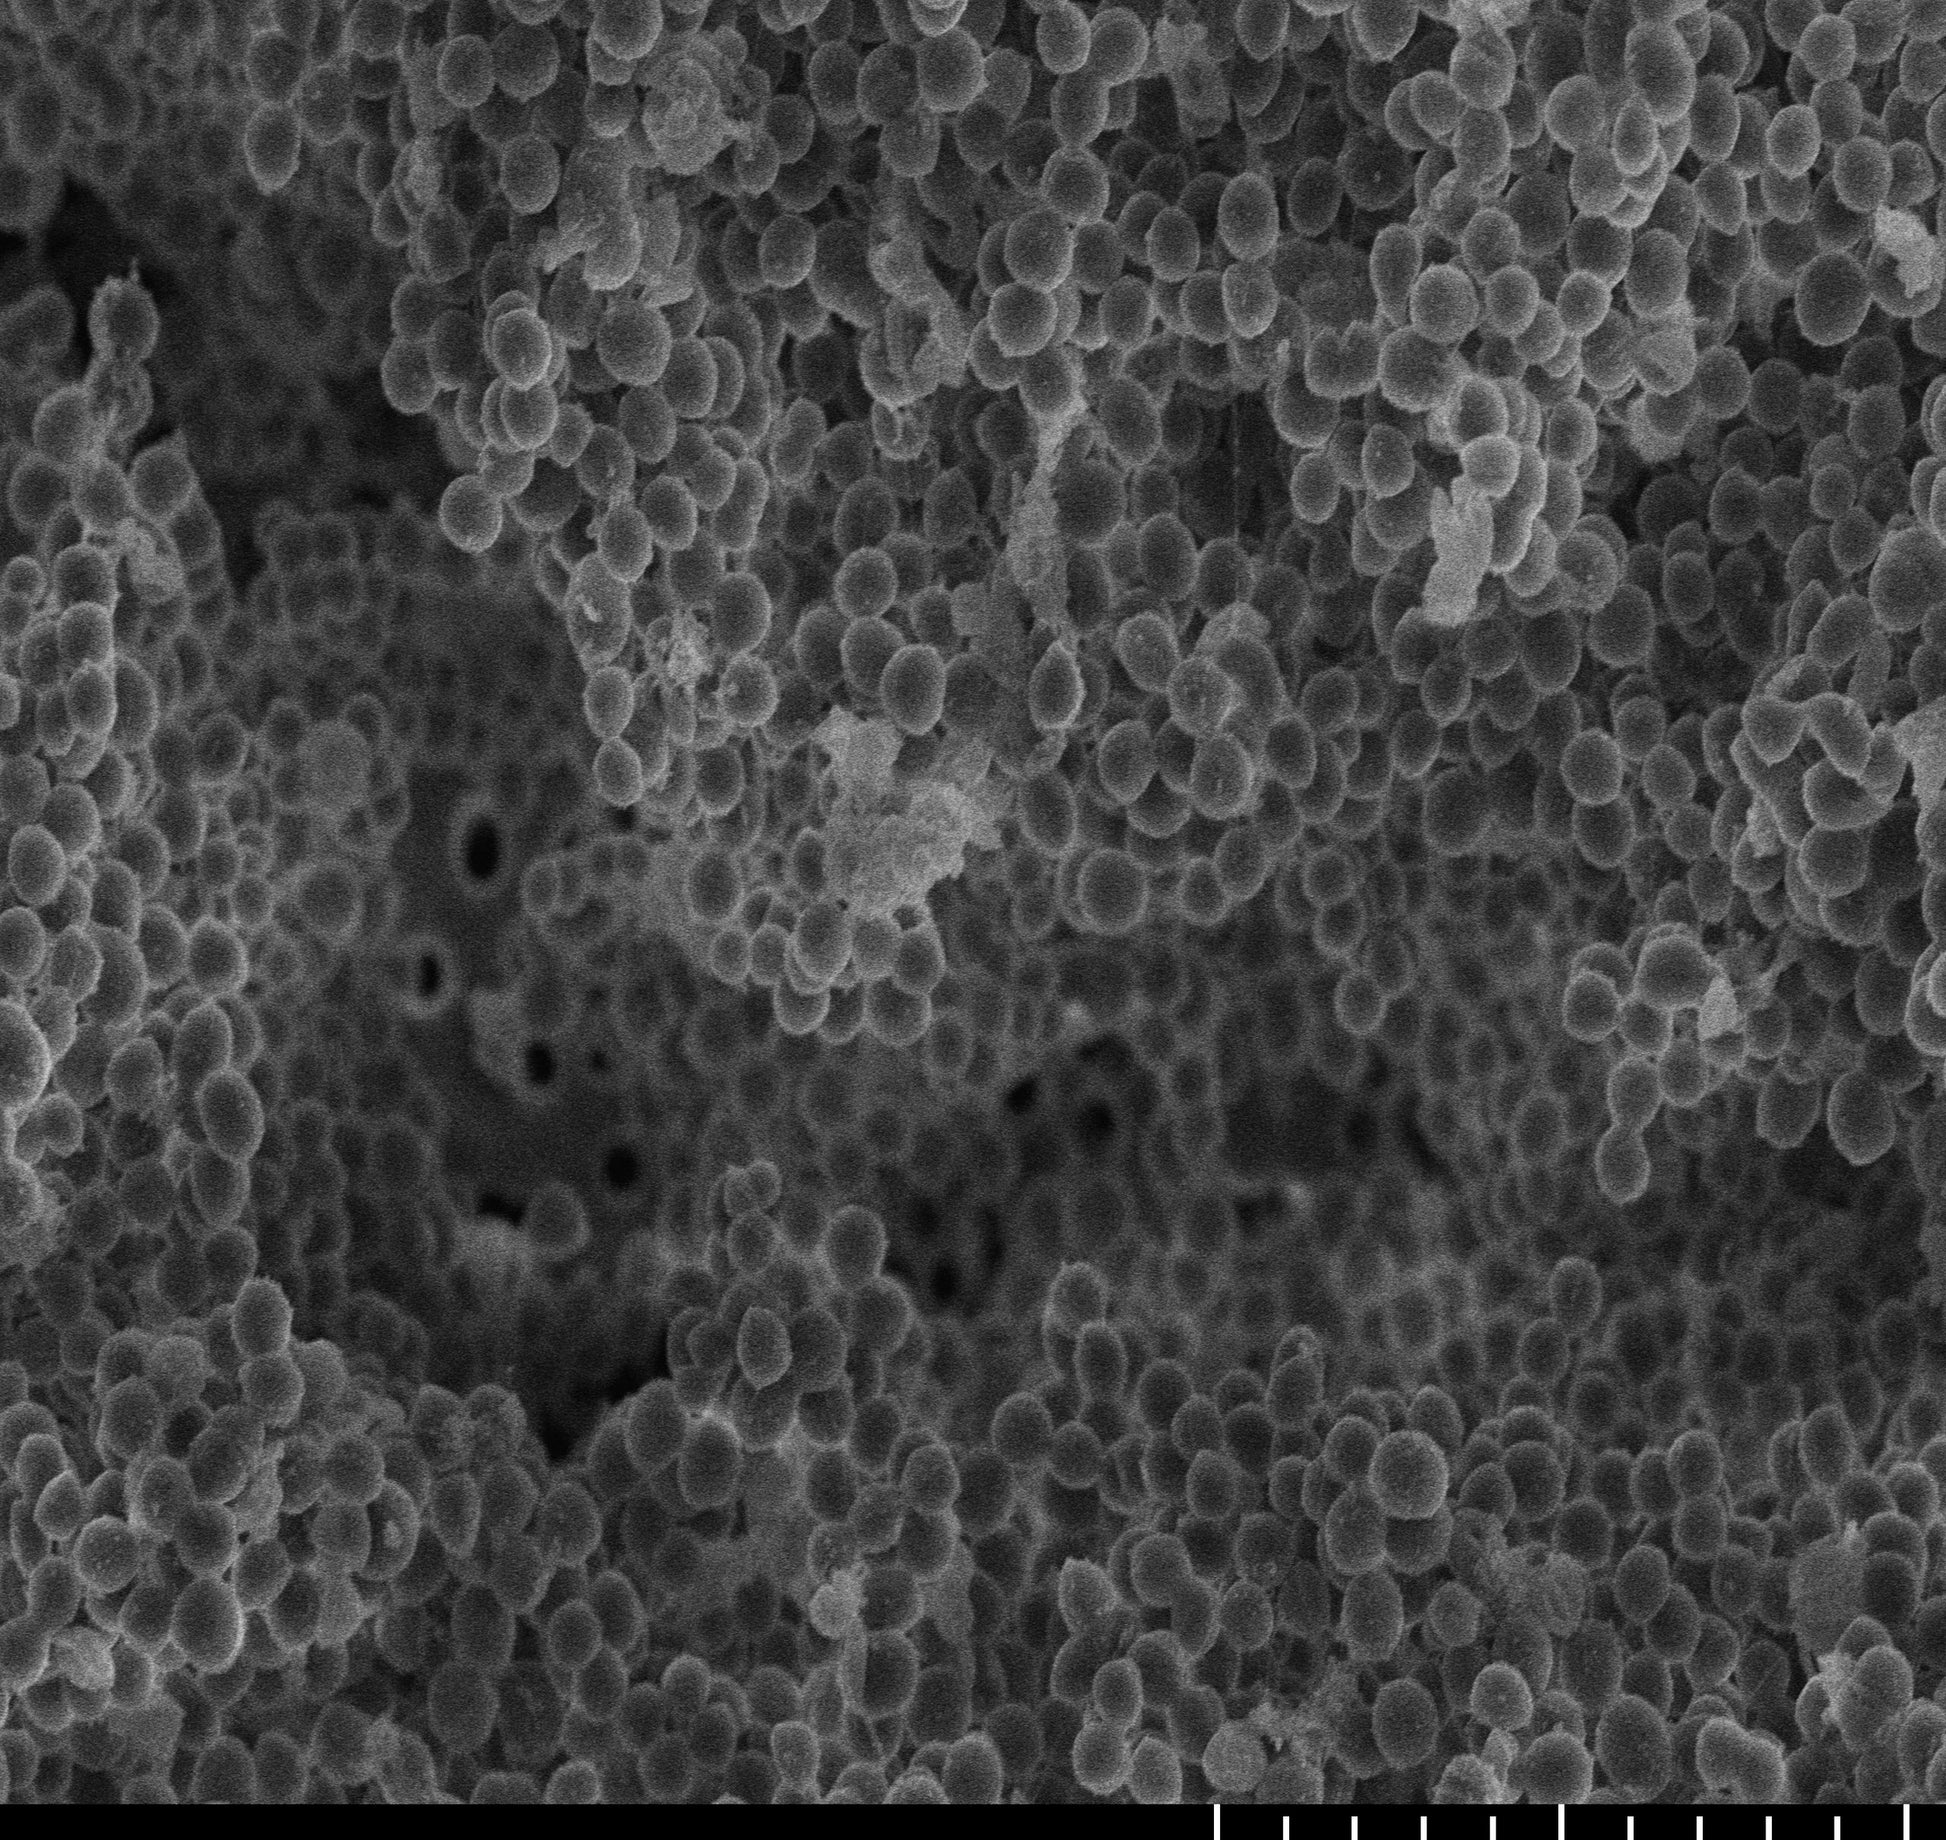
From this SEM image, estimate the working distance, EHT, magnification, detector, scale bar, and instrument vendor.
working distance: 9.4 mm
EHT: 5 kV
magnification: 4.5 K X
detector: SE(UL)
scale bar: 10000 nm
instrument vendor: Hitachi